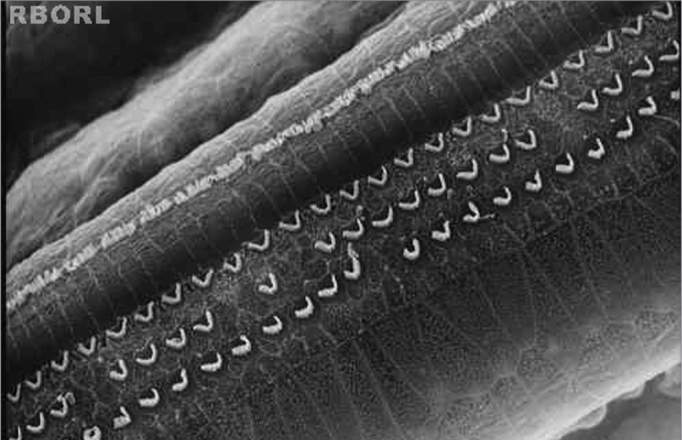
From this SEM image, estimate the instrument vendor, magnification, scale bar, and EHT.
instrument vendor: JEOL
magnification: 0.75 K X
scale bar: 10000 nm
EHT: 25 kV